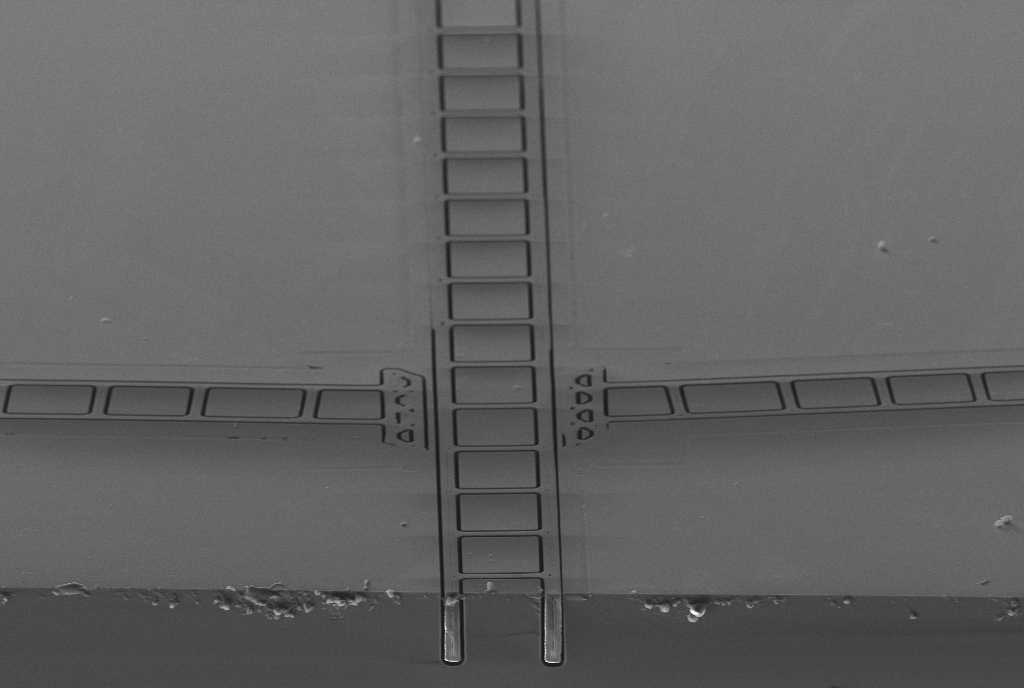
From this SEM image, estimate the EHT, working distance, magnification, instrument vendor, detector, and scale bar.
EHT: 8 kV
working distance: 7 mm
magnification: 0.46 K X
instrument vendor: Zeiss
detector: SE2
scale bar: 20000 nm